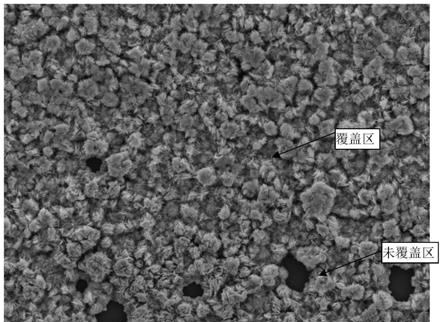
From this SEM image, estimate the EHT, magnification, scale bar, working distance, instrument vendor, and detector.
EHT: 15 kV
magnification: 1.5 K X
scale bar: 10000 nm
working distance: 10 mm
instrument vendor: JEOL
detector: LED